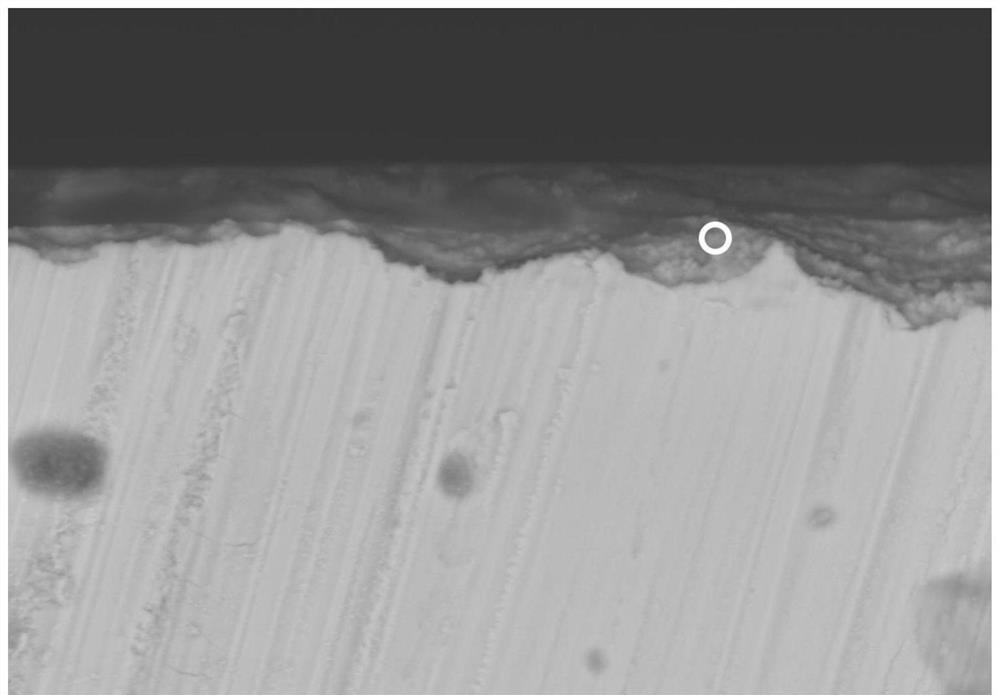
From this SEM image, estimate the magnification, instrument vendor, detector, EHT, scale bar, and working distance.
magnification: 1.5 K X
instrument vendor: Hitachi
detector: BSECOMP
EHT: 20 kV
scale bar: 30000 nm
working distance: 10.4 mm